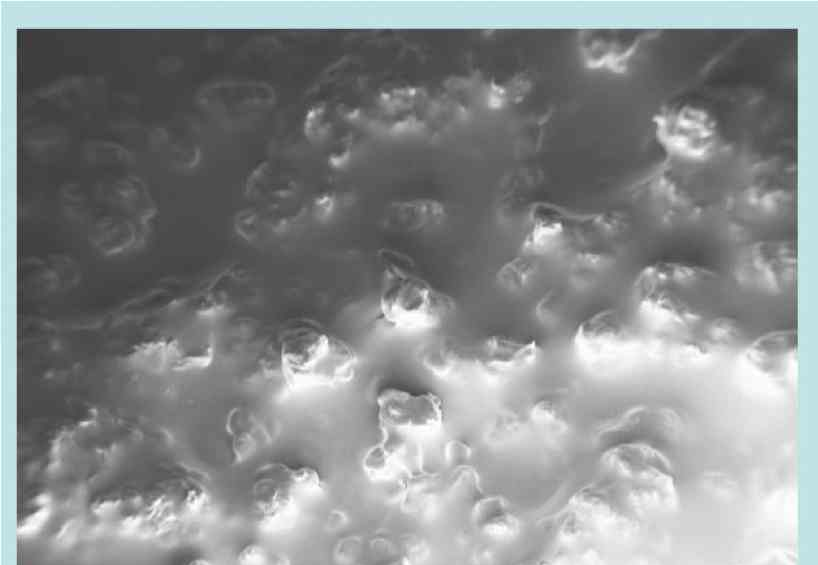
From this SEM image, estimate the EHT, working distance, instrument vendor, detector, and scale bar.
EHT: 2 kV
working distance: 8 mm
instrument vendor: Zeiss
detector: InLens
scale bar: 100000 nm